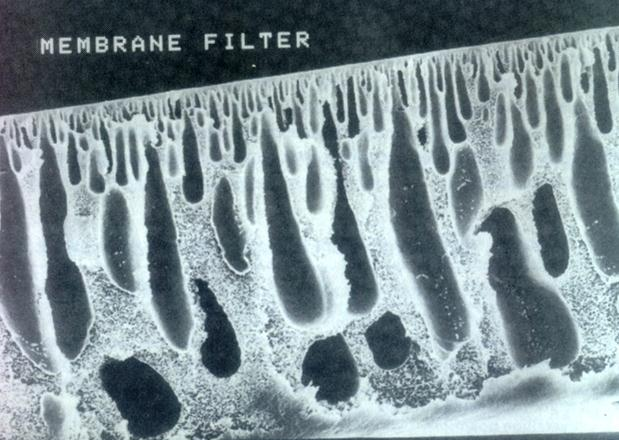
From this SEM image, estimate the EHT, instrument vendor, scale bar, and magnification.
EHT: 10 kV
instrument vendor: JEOL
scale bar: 10000 nm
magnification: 1.5 K X